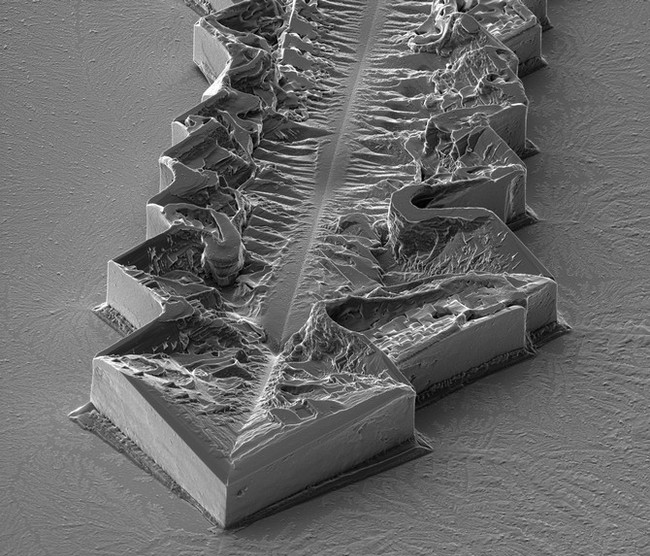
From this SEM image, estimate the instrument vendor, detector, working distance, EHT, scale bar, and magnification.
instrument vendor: FEI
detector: ETD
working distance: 6.9 mm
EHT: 5 kV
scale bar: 50000 nm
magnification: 1.636 K X